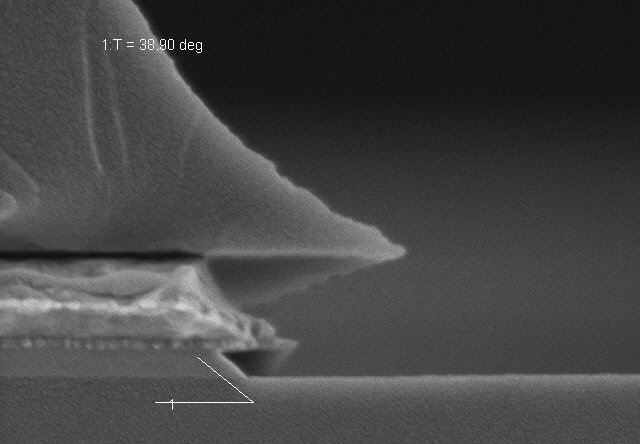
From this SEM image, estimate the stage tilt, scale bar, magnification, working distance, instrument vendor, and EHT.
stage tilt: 38.9°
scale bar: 1000 nm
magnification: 50 K X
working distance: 9.2 mm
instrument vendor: Hitachi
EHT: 3 kV